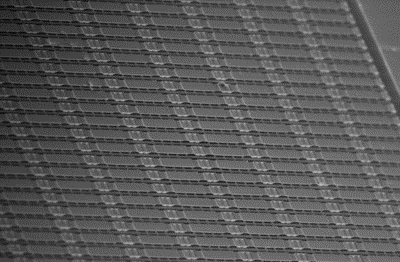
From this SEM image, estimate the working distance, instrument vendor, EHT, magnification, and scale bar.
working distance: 10 mm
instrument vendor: Hitachi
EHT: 10 kV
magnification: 0.9 K X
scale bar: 100000 nm